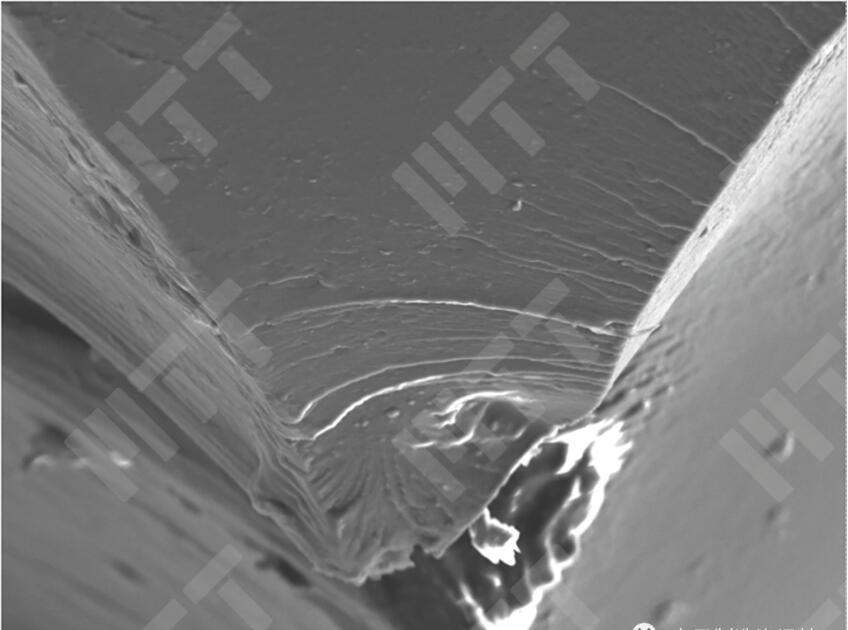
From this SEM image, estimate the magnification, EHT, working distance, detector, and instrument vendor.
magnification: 0.3 K X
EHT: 15 kV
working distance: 20 mm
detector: SE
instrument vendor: Hitachi